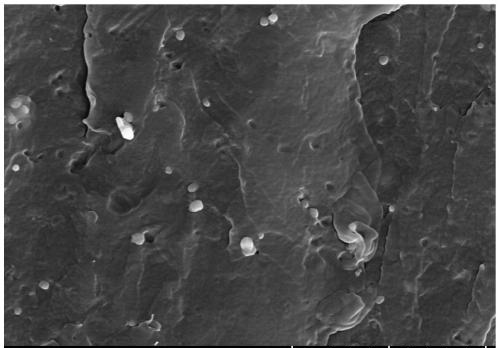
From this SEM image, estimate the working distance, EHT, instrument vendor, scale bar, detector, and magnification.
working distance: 16.3 mm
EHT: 10 kV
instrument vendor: Hitachi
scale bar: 5000 nm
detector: SE(UL)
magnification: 10 K X